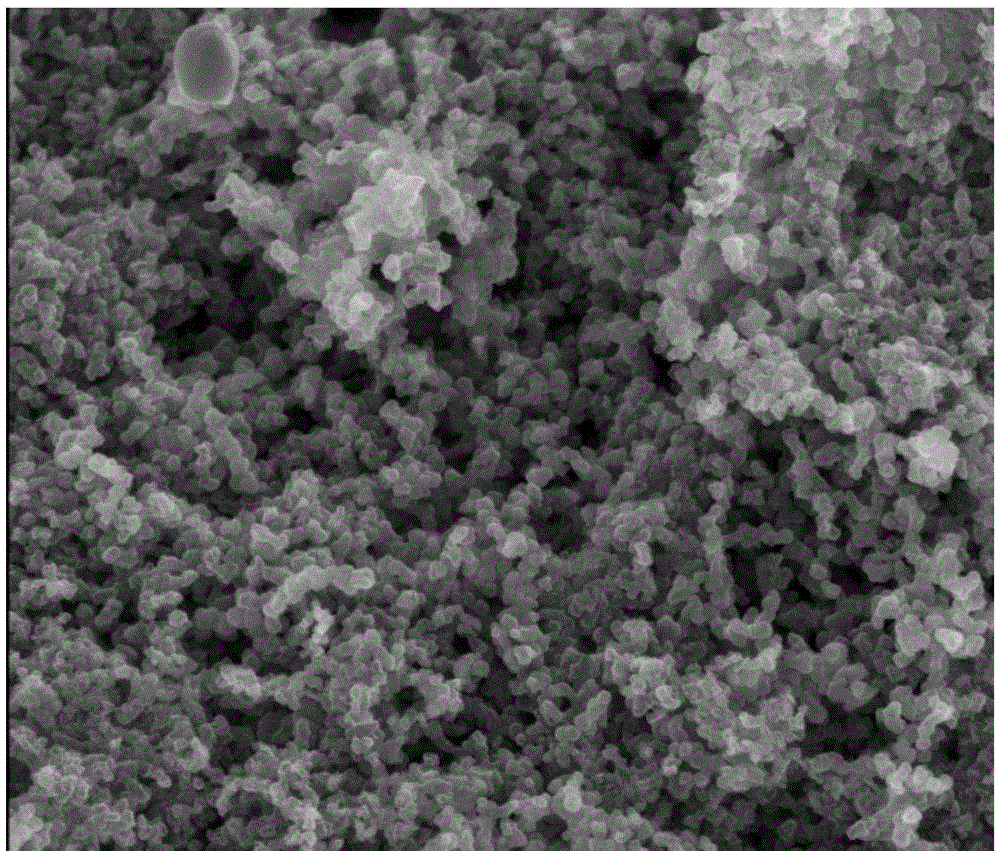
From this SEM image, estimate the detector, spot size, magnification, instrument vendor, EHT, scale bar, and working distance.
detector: TLD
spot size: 2.5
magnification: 50 K X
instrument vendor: FEI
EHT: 3 kV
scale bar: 1000 nm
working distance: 3 mm